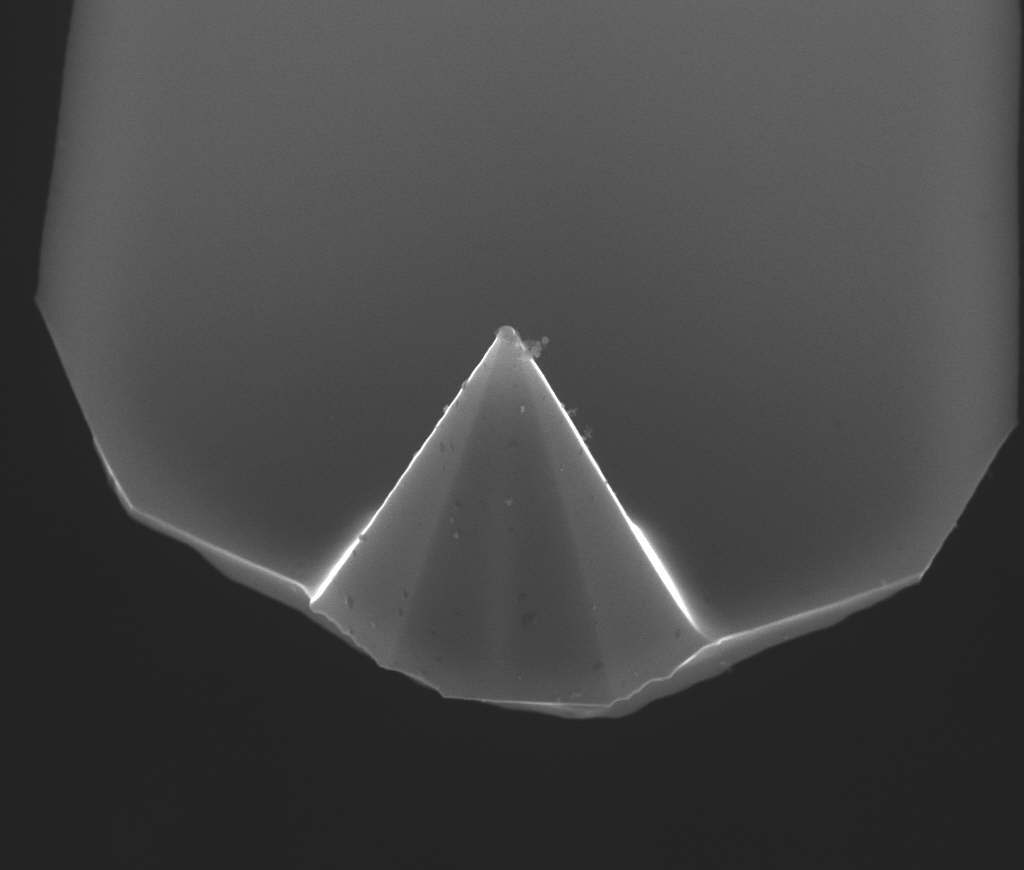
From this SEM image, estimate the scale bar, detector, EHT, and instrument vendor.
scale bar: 5000 nm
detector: HELIX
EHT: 15 kV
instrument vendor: FEI